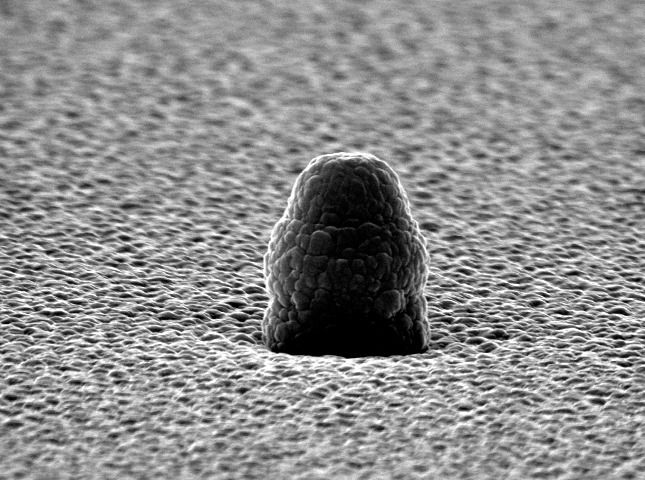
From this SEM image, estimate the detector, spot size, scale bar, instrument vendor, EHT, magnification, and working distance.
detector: SE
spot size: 3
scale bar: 1000 nm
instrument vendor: FEI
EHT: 25 kV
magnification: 26.769 K X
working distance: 7.6 mm